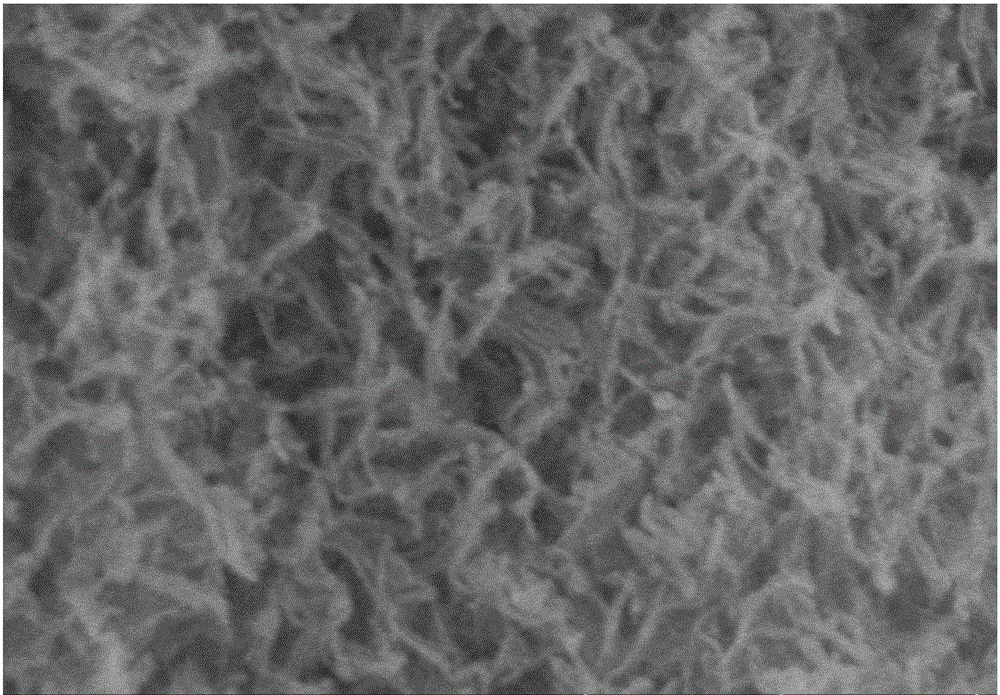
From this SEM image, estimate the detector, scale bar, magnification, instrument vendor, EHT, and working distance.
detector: SE(U)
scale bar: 500 nm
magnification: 100 K X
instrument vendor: Hitachi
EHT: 10 kV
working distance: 13.5 mm